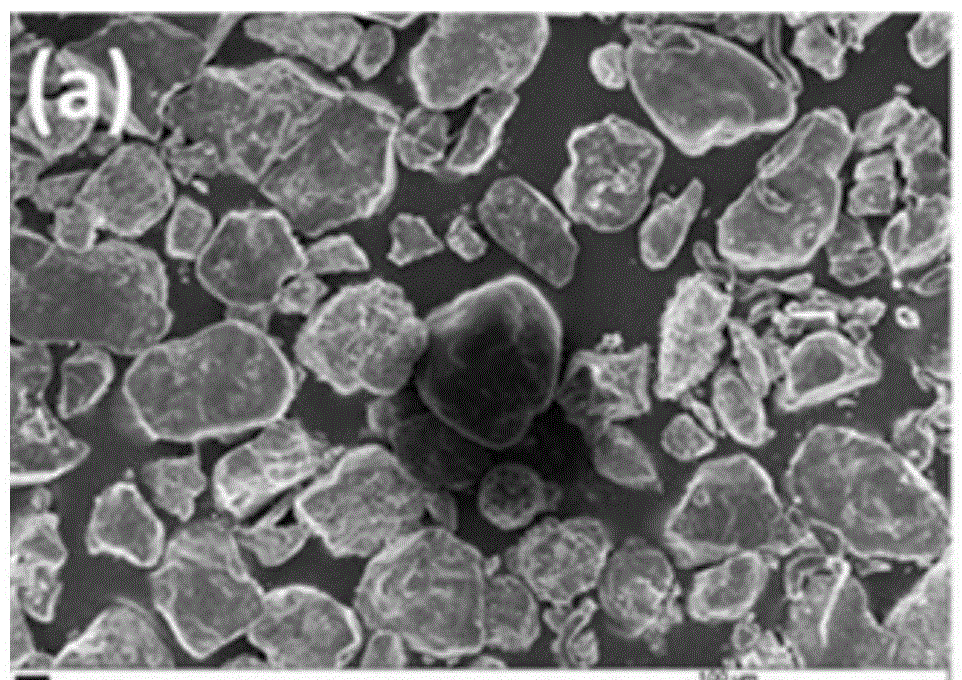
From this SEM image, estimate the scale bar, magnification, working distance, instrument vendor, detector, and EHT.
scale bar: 100000 nm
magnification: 0.1 K X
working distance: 6 mm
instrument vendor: Zeiss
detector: InLens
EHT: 20 kV